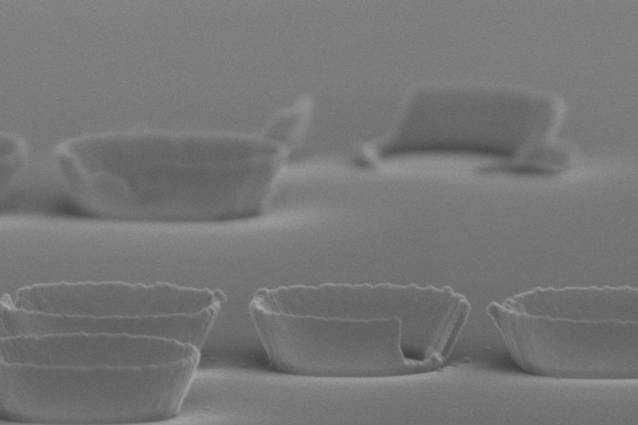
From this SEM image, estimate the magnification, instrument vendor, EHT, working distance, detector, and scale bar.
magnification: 50 K X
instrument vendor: Hitachi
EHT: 15 kV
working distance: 14.2 mm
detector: SE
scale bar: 1000 nm